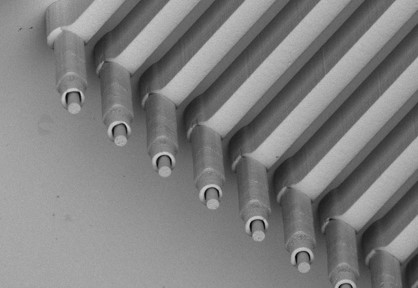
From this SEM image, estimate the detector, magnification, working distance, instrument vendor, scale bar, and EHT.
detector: BSE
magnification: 0.15 K X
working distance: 13.1 mm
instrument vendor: Hitachi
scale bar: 300000 nm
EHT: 15 kV